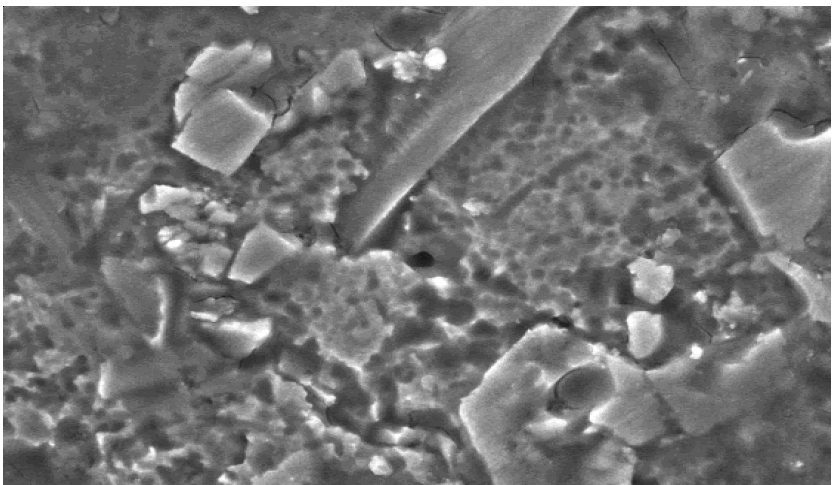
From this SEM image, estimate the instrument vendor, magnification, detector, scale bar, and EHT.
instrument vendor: JEOL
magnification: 3.5 K X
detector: SEI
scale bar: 5000 nm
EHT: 10 kV